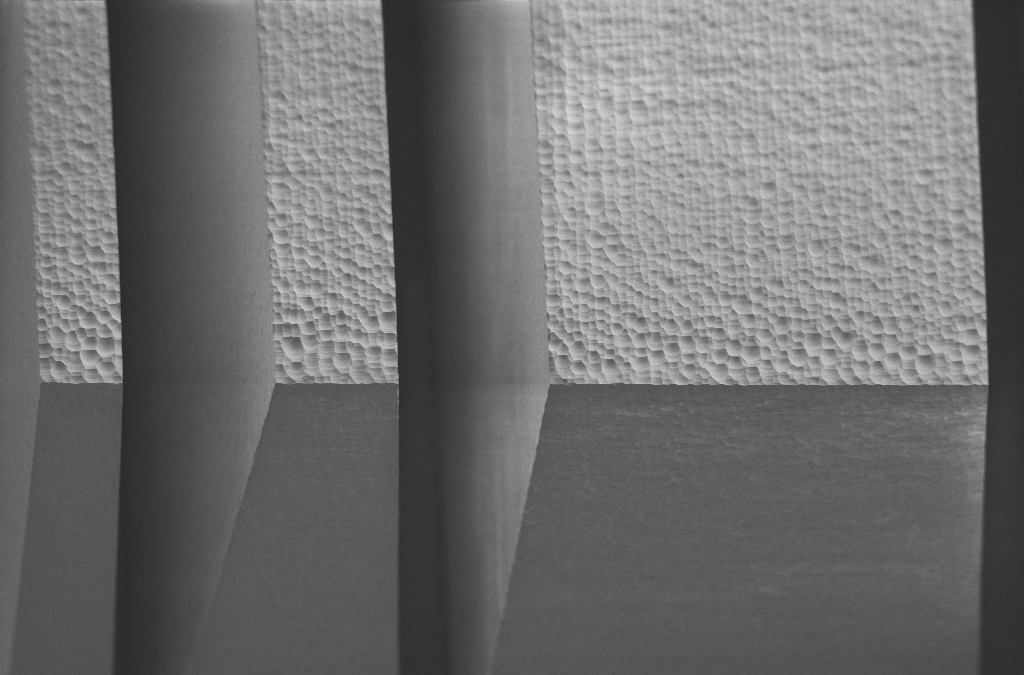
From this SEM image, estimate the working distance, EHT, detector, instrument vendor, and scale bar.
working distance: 6.6 mm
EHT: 2 kV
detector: SE2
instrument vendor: Zeiss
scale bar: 20000 nm